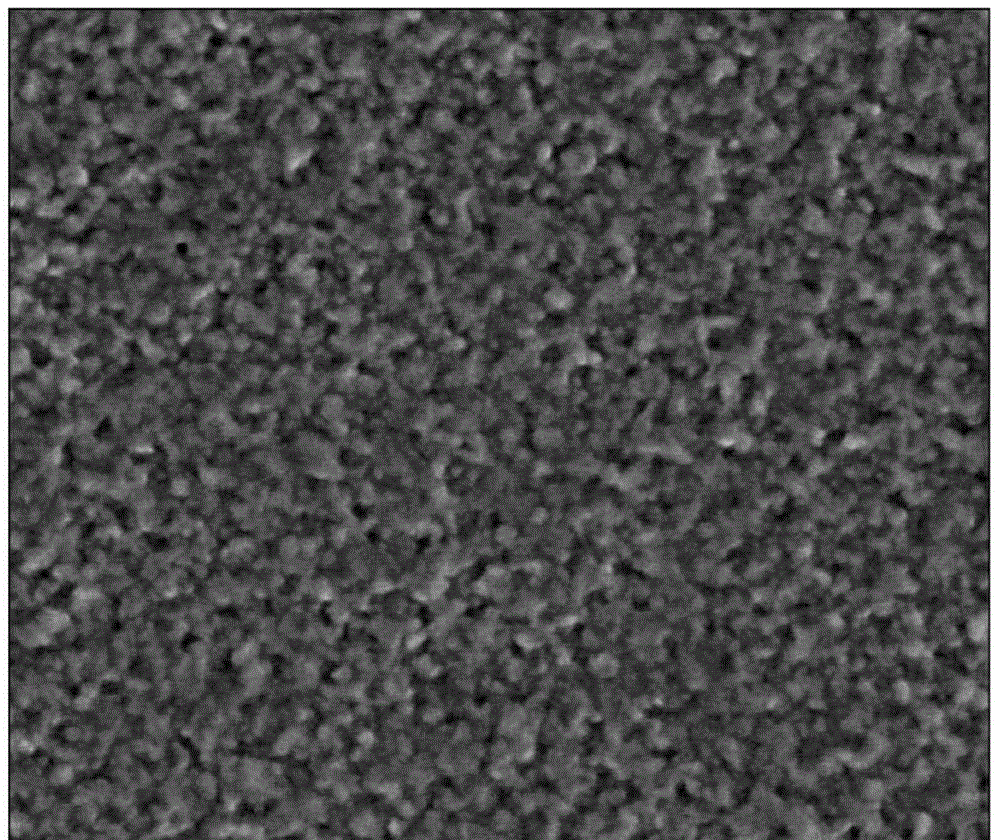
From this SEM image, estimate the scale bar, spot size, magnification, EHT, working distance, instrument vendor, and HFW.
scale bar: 1000 nm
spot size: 4.5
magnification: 50 K X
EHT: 5 kV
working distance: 10 mm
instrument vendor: FEI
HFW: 2.98 µm